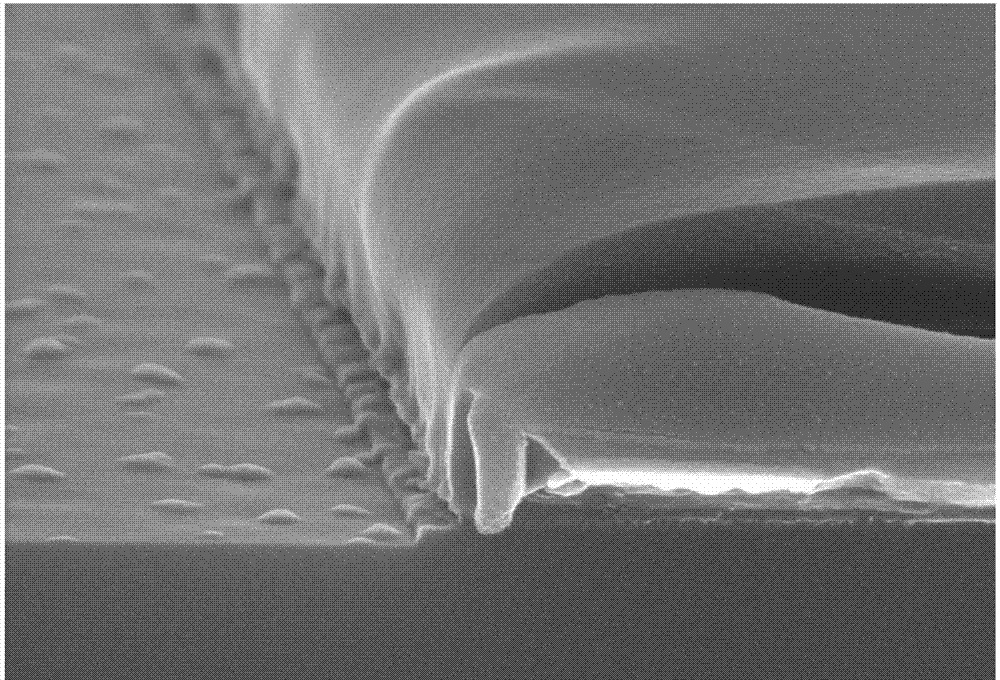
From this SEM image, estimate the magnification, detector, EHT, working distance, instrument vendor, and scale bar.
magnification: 50 K X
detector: SE(U)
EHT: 10 kV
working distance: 10.4 mm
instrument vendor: Hitachi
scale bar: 1000 nm